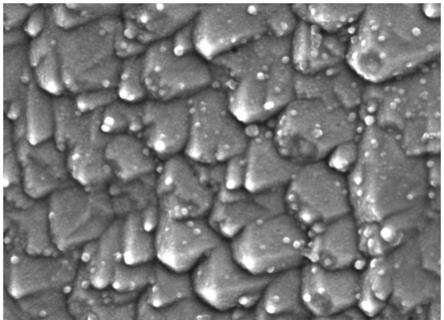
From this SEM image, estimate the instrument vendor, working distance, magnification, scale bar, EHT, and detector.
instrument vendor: JEOL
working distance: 10.4 mm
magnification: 30 K X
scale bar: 100 nm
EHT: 5 kV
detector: LED